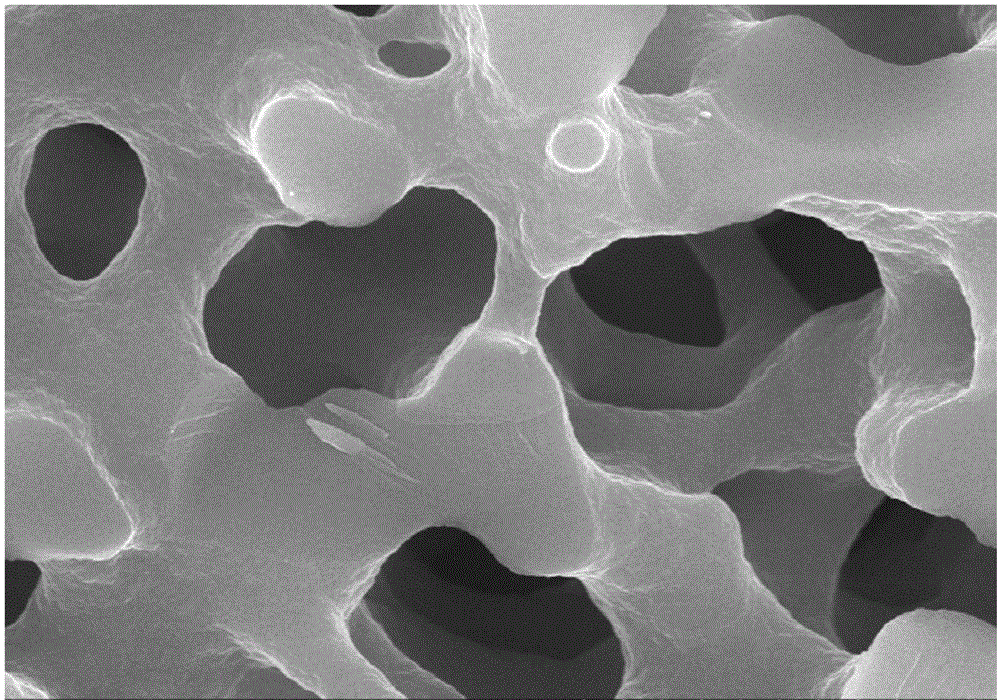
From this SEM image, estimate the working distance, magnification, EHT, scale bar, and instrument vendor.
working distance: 9.6 mm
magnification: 10 K X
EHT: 20 kV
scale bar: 5000 nm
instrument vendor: Hitachi